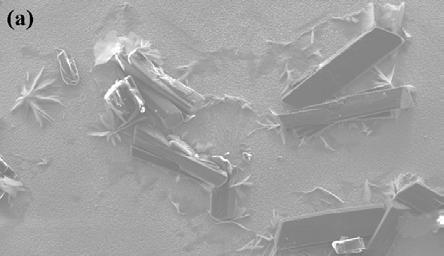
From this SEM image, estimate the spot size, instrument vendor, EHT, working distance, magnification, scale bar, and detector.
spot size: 4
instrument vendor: FEI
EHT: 20 kV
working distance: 7.8 mm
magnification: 0.1 K X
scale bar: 200000 nm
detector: SE